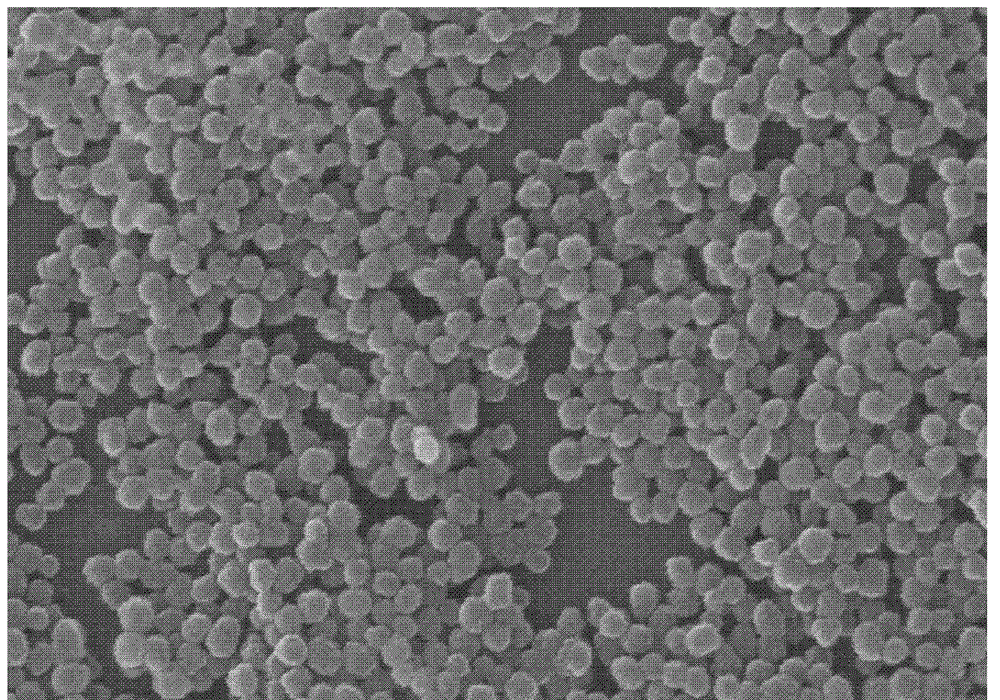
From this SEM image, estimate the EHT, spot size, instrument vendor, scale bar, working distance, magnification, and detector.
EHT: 20 kV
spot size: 3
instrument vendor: FEI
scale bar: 1000 nm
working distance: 7.4 mm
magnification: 20 K X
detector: SE